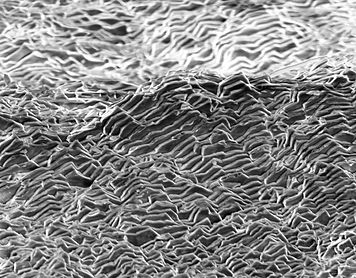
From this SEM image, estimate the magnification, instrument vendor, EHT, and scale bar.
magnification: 1.3 K X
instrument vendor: JEOL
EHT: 10 kV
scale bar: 20000 nm